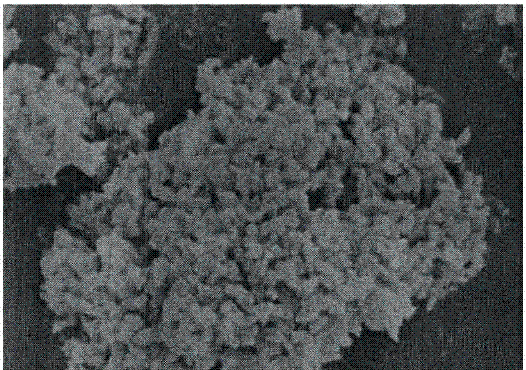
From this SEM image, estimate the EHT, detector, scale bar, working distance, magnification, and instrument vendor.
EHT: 10 kV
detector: SE(M)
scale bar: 1000 nm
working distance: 8.7 mm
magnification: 15 K X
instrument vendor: Hitachi